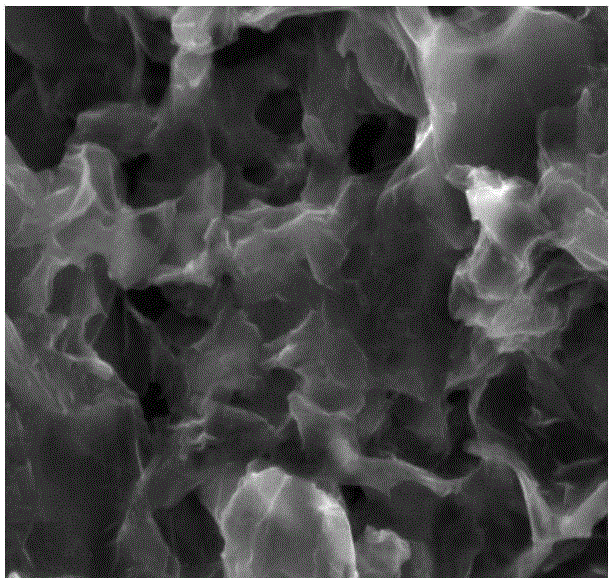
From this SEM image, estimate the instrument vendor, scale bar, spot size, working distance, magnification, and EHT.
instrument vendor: FEI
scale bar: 10000 nm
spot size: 4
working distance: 10.7 mm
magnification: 5 K X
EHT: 20 kV